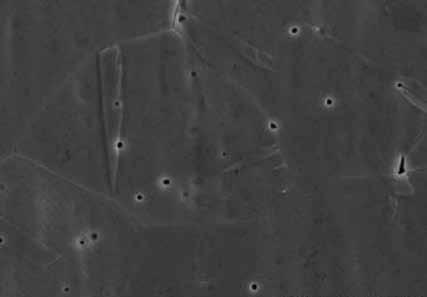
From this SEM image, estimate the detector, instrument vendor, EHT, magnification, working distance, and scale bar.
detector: SE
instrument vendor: Hitachi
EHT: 25 kV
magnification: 2 K X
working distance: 8.1 mm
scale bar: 20000 nm